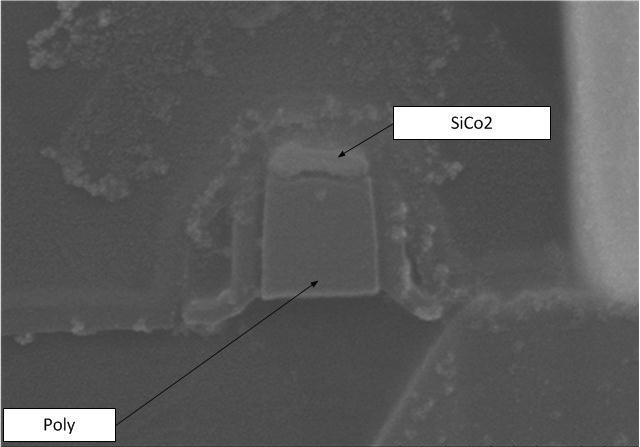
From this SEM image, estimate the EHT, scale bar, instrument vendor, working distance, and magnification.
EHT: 10 kV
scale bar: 300 nm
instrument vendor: Hitachi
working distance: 8.1 mm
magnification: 150 K X